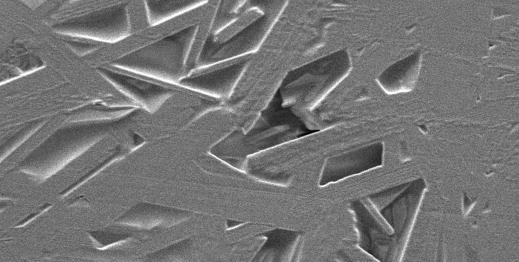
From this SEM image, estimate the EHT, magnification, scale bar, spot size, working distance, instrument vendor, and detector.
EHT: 5 kV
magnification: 3.5 K X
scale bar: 10000 nm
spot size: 3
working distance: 7 mm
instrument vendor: FEI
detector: SE